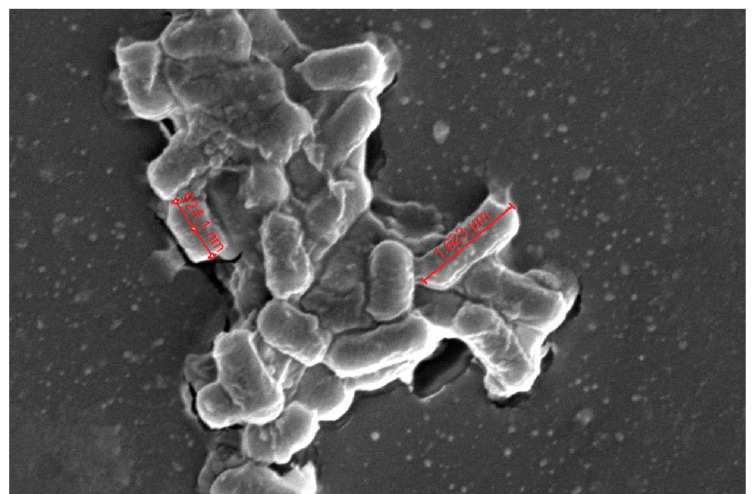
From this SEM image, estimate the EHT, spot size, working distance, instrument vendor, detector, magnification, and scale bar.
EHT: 5 kV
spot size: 3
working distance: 5.4 mm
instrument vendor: FEI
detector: ETD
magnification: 40 K X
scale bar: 3000 nm